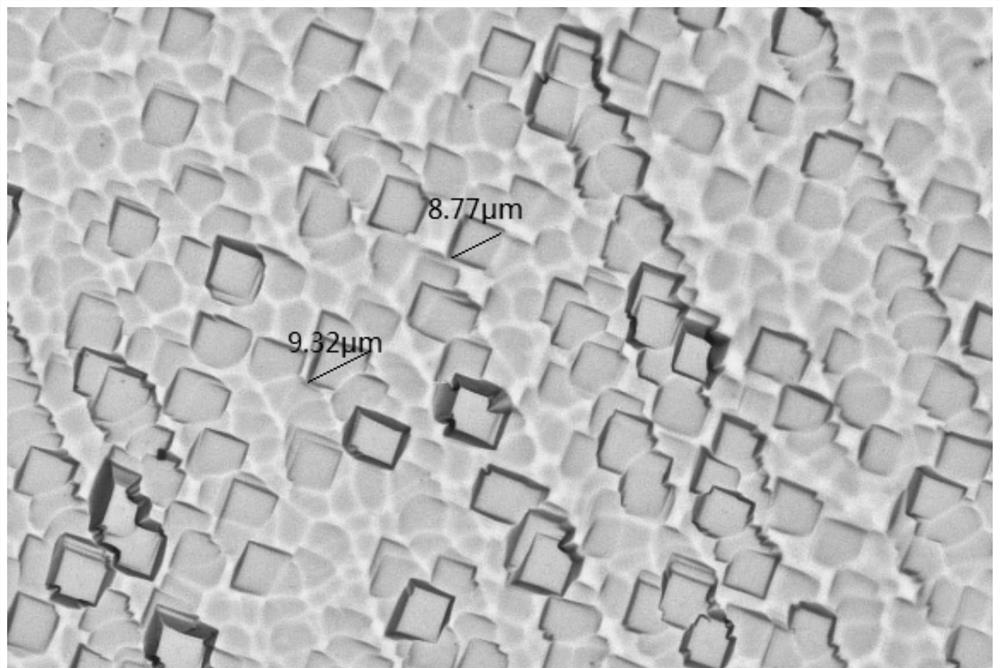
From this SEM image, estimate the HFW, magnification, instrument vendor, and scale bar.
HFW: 177 µm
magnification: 0.05 K X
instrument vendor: other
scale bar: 15000 nm